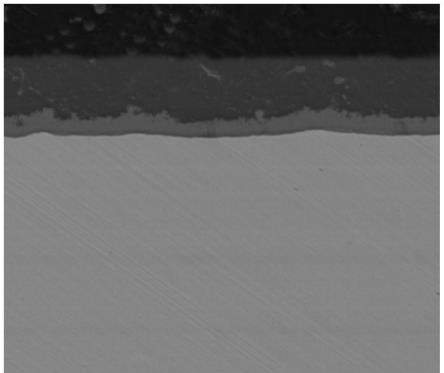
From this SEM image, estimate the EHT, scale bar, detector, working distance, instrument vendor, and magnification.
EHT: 20 kV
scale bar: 50000 nm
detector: BSED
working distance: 8.8 mm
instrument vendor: FEI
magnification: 1 K X